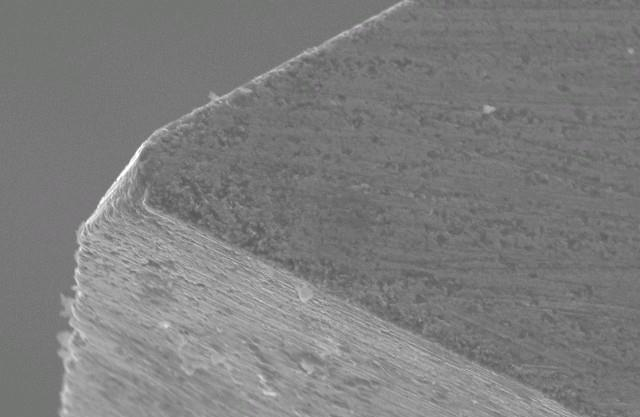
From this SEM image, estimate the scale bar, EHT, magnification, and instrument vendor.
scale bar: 10000 nm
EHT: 20 kV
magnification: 1 K X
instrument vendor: JEOL